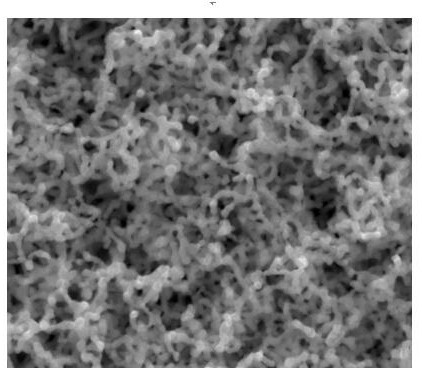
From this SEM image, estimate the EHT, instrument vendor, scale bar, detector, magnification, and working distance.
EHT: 10 kV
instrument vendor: FEI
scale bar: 500 nm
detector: ETD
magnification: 200 K X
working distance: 9.1 mm